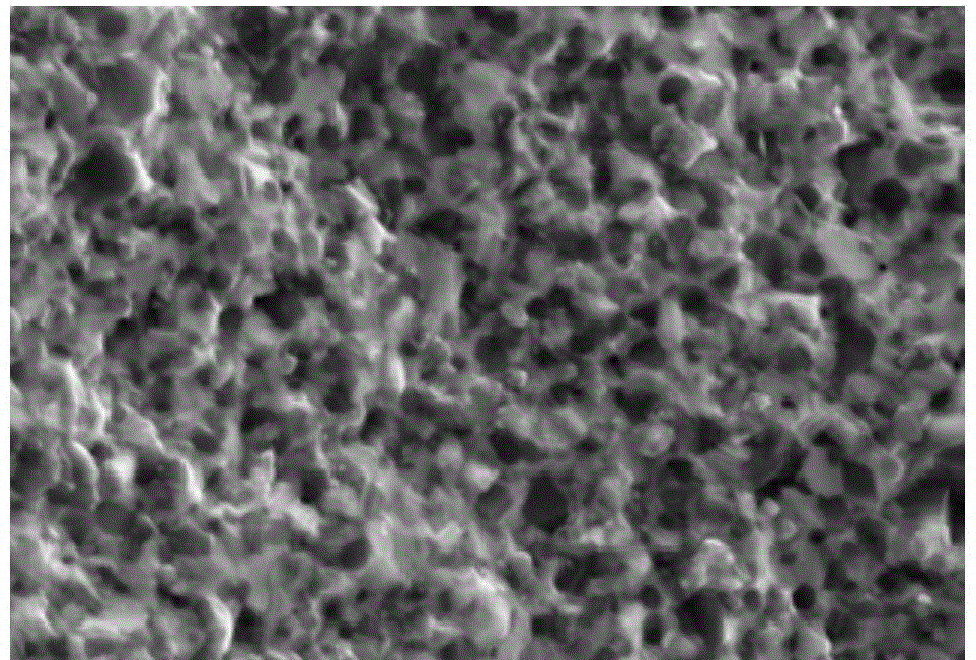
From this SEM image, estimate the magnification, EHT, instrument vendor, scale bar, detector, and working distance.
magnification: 50 K X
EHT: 15 kV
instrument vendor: Hitachi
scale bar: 1000 nm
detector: SE(M)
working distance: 15.8 mm